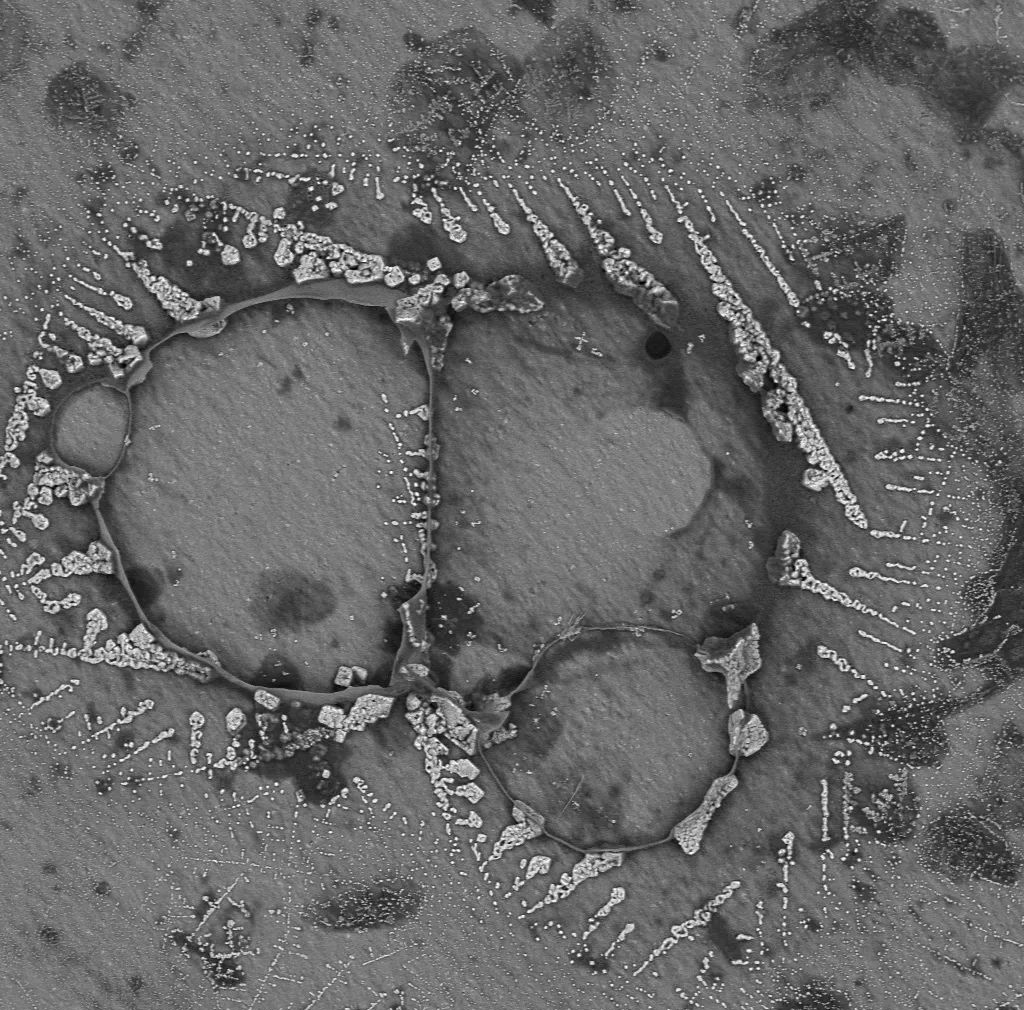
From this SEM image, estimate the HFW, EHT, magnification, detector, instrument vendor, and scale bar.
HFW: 757 µm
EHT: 15 kV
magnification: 0.35 K X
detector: BSD Full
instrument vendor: other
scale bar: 200000 nm